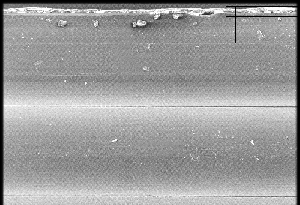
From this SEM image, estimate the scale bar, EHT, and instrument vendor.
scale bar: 300000 nm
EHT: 10 kV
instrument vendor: JEOL